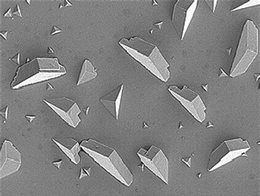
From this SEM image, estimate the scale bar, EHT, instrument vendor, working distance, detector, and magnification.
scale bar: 1000 nm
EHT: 3 kV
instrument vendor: JEOL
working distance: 9 mm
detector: SEI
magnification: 3 K X